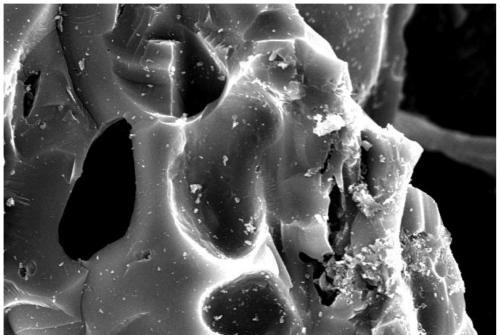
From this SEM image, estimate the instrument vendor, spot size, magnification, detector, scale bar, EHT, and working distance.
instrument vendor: JEOL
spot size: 50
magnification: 1 K X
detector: SEI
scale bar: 10000 nm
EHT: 20 kV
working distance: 21 mm